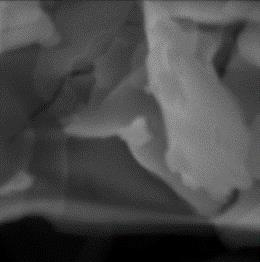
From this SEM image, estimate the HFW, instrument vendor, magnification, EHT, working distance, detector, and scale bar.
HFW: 9.685 µm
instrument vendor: Tescan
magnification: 15 K X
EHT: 15 kV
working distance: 15.97 mm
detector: SE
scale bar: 2000 nm